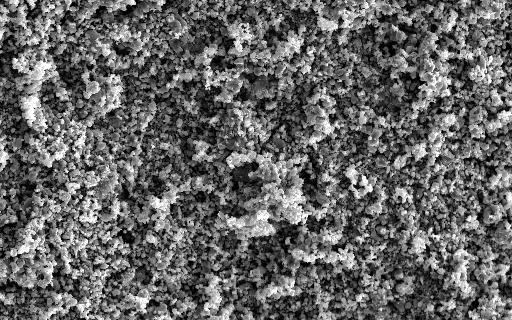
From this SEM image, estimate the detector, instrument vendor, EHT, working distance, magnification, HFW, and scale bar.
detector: SE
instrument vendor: Tescan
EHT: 20 kV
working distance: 10.27 mm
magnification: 1 K X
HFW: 144 µm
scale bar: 20000 nm